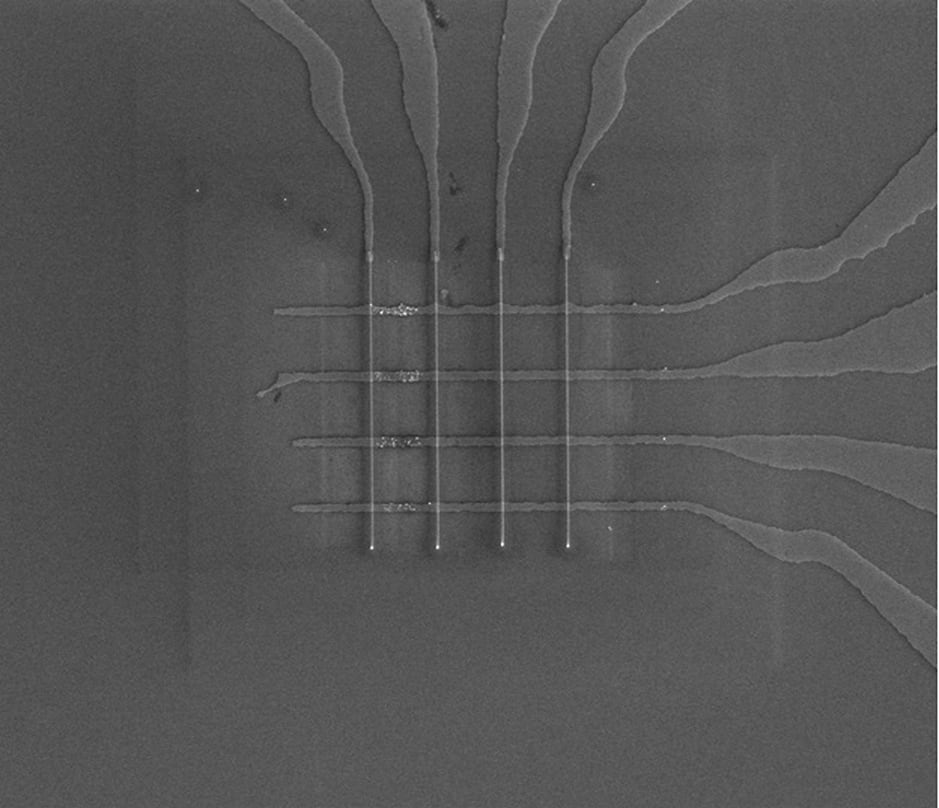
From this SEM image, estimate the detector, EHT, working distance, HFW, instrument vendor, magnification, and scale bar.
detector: ETD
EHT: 10 kV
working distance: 5.1 mm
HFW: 102 µm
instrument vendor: FEI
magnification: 2.5 K X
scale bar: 30000 nm